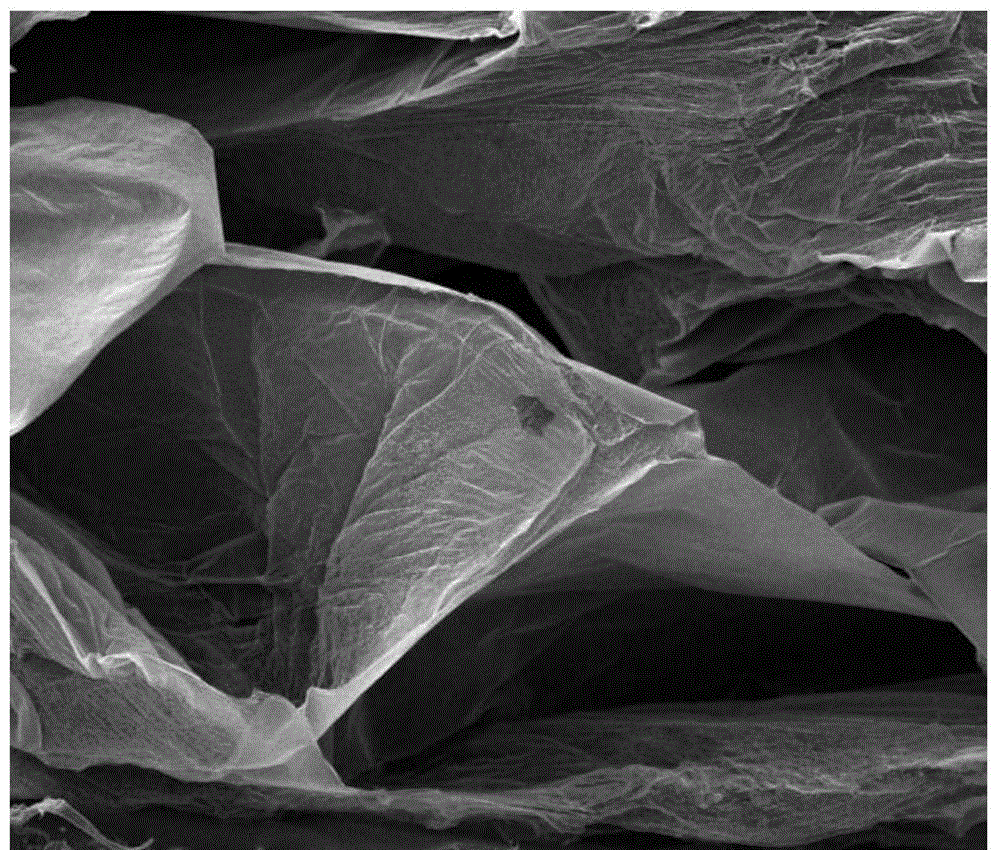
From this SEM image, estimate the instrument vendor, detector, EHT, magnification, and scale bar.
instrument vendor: FEI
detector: ETD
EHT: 10 kV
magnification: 0.2 K X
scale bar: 400000 nm